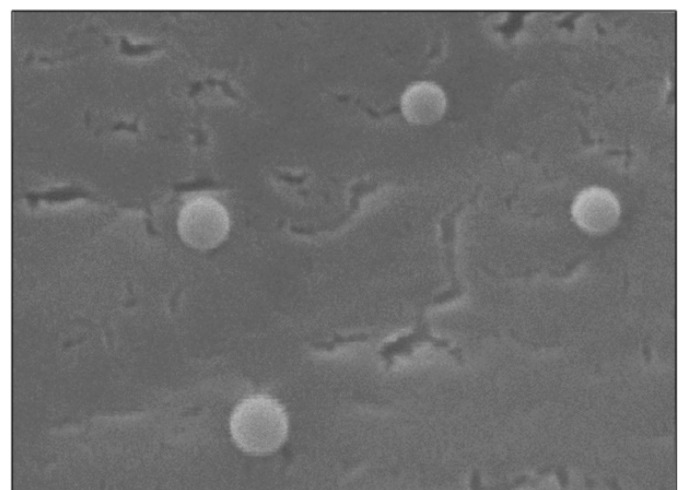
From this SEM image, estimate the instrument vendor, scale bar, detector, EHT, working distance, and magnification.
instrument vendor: JEOL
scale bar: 100 nm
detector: SEI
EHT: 5 kV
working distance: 5.7 mm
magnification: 100 K X